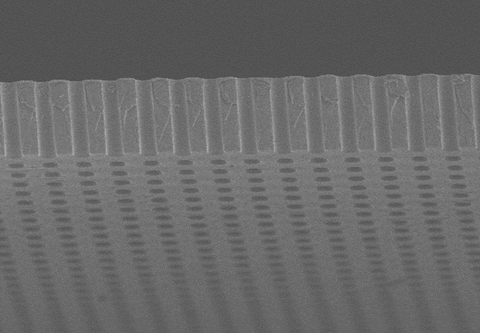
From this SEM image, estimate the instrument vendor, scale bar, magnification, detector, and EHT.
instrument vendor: Hitachi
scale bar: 30000 nm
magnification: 1.5 K X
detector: SE(M)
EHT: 3 kV